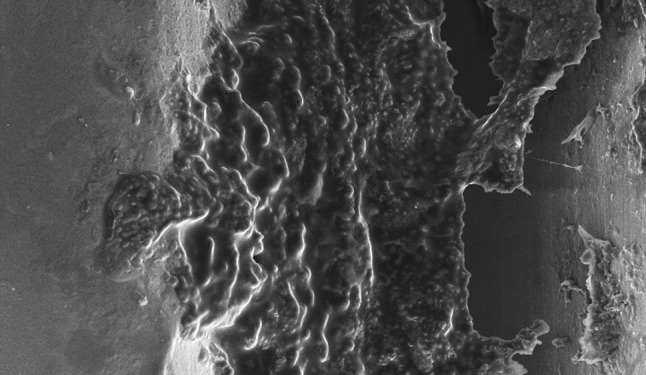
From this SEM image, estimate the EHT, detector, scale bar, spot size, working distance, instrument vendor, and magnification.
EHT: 15 kV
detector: SE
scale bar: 100000 nm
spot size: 2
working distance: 10 mm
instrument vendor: FEI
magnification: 0.25 K X